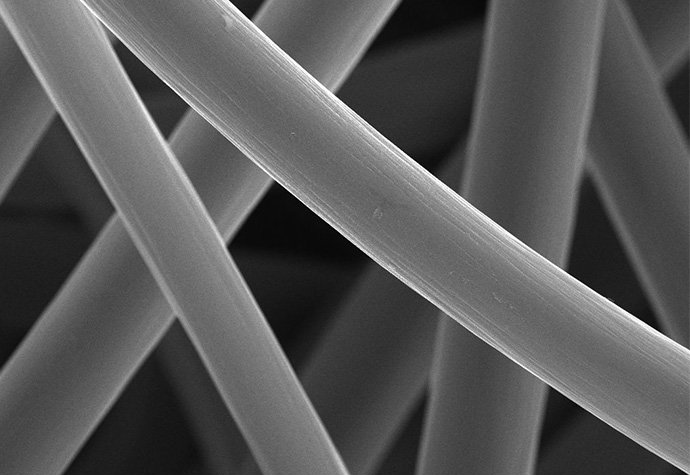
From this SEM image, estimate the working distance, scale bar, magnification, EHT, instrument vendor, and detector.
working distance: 10.5 mm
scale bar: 20000 nm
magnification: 5 K X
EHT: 25 kV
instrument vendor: FEI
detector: SE